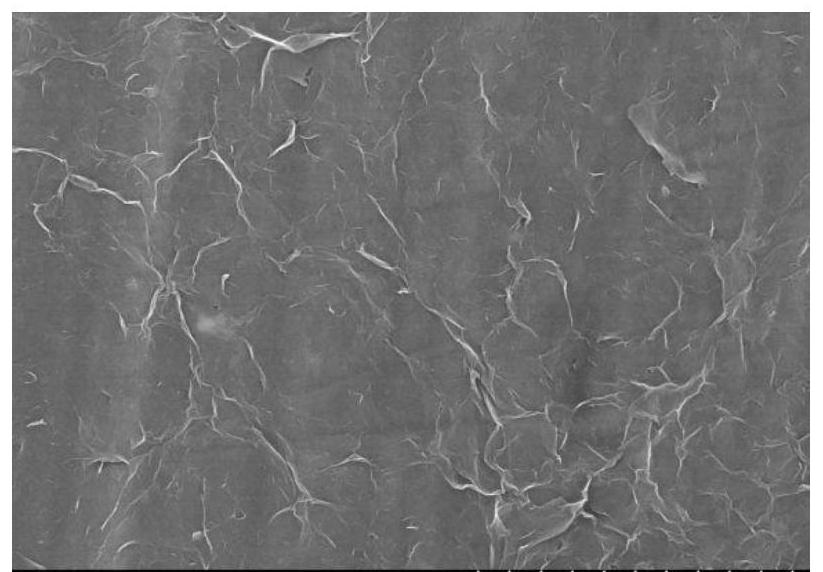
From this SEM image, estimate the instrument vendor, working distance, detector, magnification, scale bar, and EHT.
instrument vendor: Hitachi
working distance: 13.2 mm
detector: SE(M)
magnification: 5 K X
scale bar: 10000 nm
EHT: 20 kV